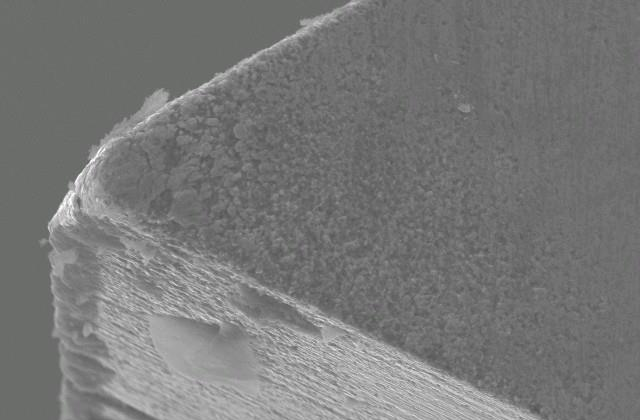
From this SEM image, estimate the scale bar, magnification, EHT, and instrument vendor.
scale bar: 10000 nm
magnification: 1 K X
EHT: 20 kV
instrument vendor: JEOL